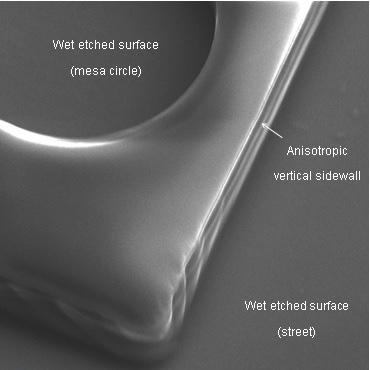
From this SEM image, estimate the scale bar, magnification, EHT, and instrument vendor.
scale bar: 2000 nm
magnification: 4.28 K X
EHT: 10 kV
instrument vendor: Zeiss